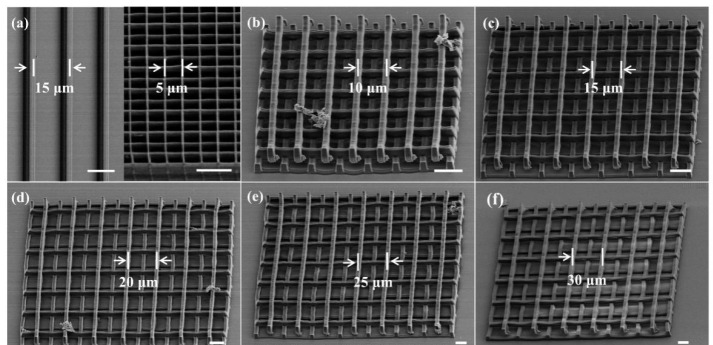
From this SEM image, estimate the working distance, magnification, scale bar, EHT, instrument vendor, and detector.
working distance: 15.8 mm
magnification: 0.6 K X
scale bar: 50000 nm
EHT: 5 kV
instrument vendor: Hitachi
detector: SE(M)